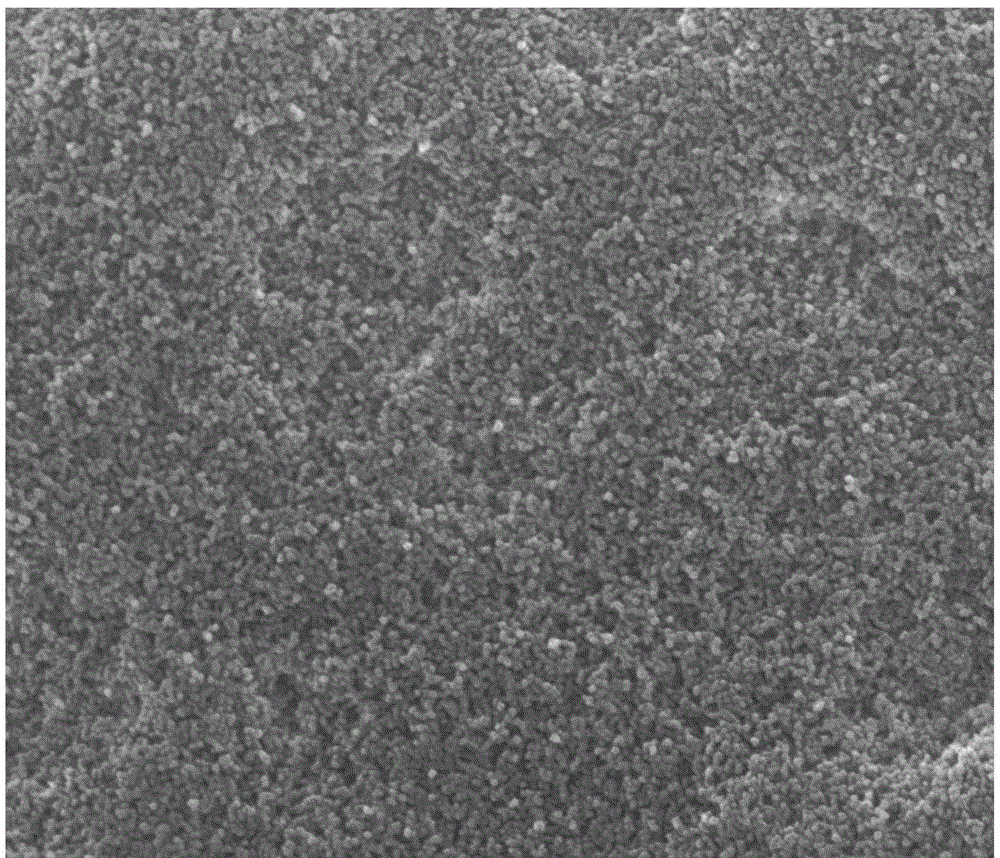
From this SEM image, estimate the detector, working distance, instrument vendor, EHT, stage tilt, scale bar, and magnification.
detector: SE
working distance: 9.8 mm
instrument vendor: FEI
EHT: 30 kV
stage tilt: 0°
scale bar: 1000 nm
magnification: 50 K X